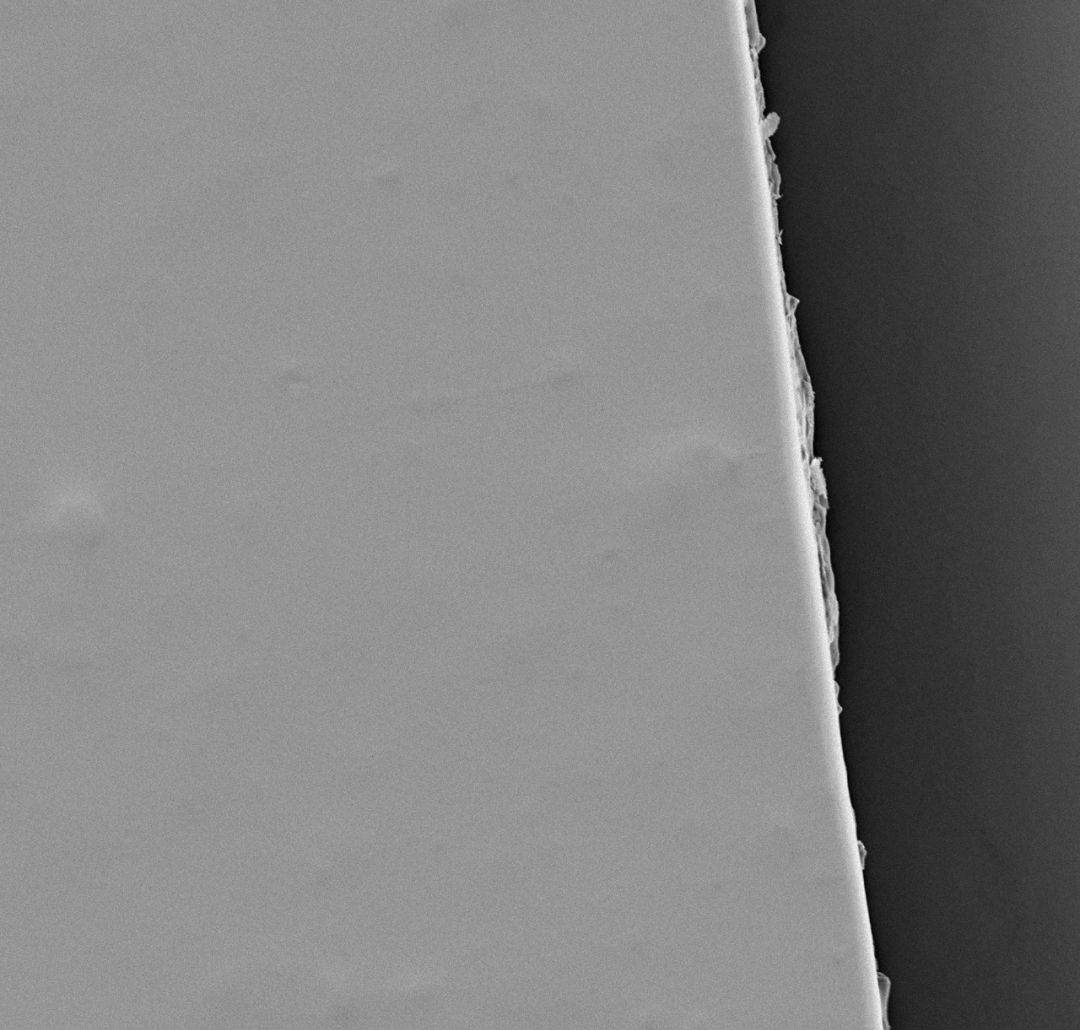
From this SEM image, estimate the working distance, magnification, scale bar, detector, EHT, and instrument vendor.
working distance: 9.1 mm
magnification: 1 K X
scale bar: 50000 nm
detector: Mix 50%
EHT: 10 kV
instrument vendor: other